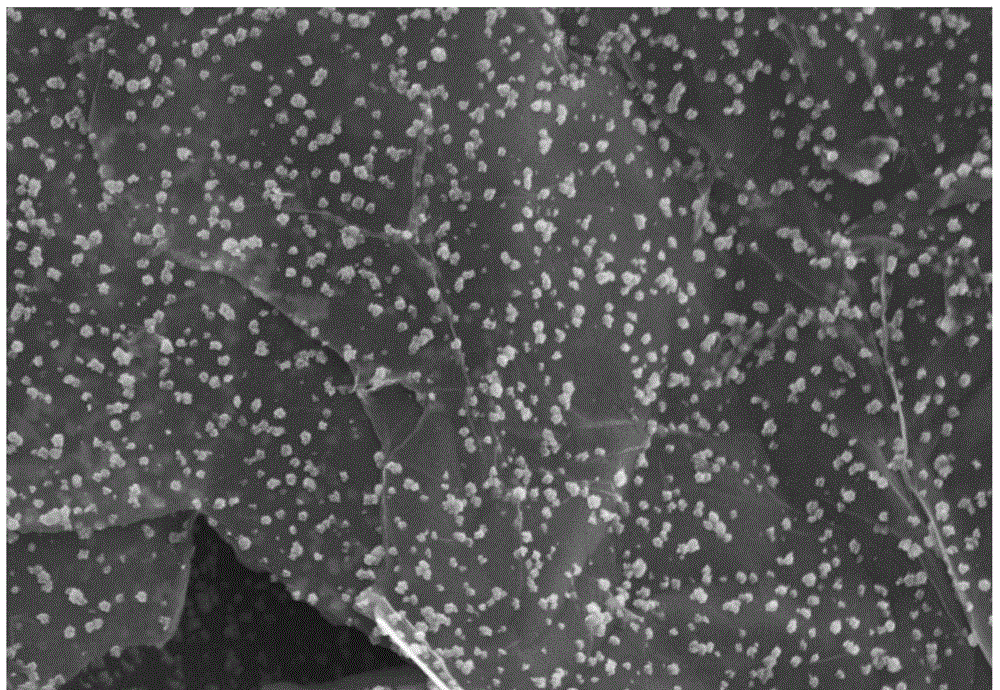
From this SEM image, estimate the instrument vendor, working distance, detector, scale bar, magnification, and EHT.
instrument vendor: Zeiss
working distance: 8 mm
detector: InLens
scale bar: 100 nm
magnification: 30 K X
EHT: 5 kV